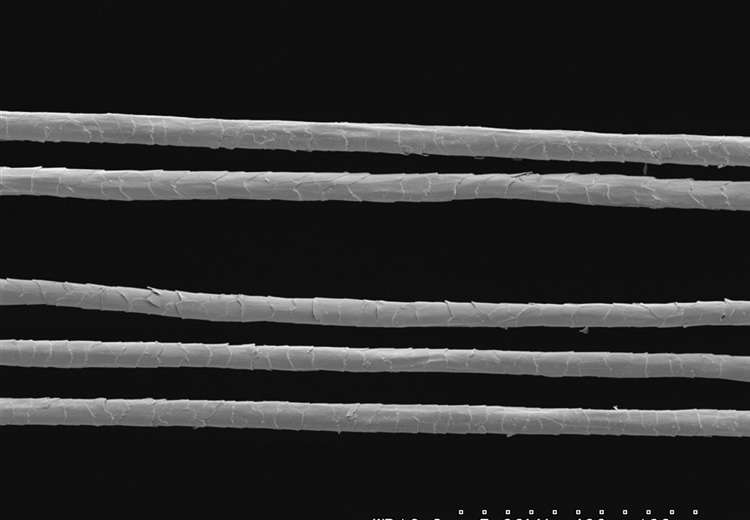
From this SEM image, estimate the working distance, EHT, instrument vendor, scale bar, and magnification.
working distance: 16.3 mm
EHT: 5 kV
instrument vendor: Hitachi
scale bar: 100000 nm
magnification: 0.4 K X